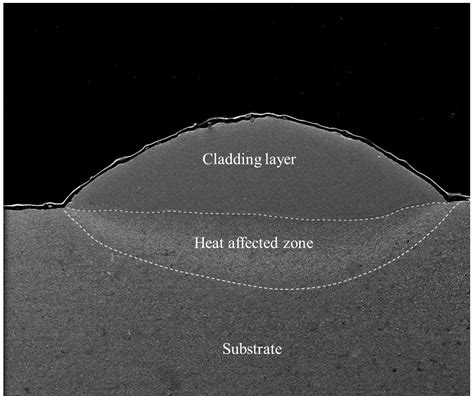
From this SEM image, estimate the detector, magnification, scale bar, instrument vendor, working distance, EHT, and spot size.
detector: ETD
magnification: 0.09 K X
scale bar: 500000 nm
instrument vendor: FEI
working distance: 11.4 mm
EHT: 15 kV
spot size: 4.5